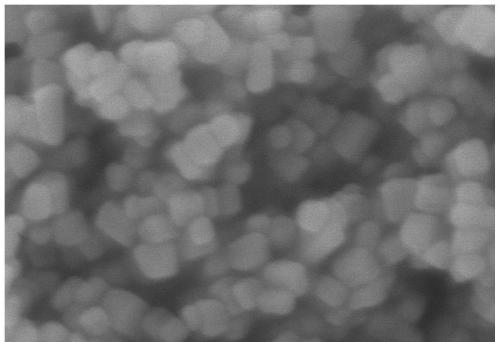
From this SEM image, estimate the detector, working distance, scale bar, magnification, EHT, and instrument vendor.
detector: SE(UL)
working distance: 8.2 mm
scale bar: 200 nm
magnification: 200 K X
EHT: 5 kV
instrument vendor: Hitachi